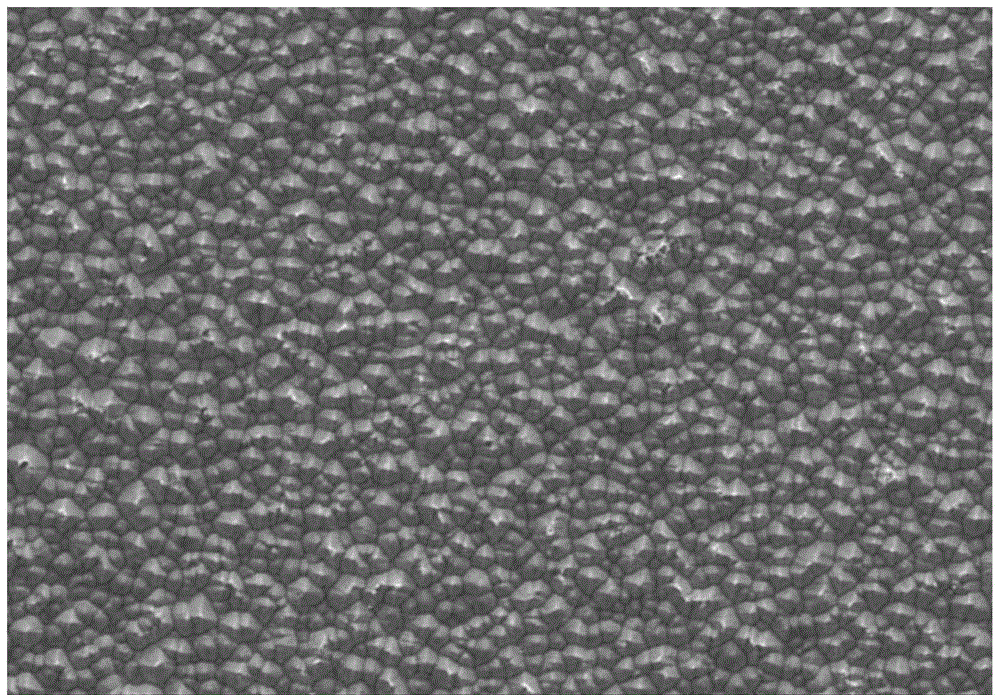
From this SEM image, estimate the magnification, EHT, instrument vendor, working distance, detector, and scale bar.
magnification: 0.4 K X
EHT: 10 kV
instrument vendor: Hitachi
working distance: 9.2 mm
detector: SE(M)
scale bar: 100000 nm